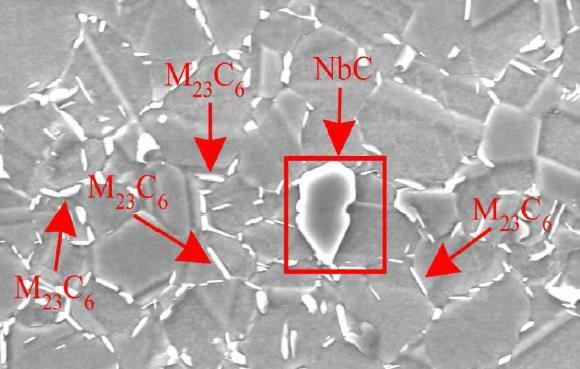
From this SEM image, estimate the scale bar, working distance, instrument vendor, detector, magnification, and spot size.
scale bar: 10000 nm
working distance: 10.6 mm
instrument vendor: FEI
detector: SE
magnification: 2.5 K X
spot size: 5